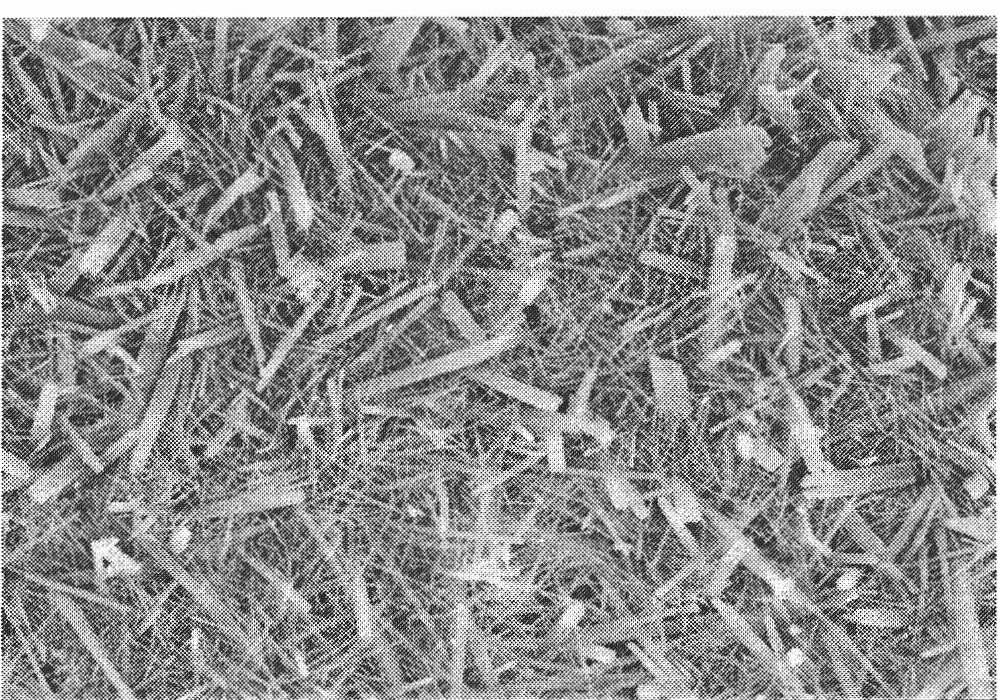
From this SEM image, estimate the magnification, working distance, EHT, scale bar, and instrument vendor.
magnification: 15 K X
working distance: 8.2 mm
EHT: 5 kV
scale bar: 3000 nm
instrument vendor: Hitachi